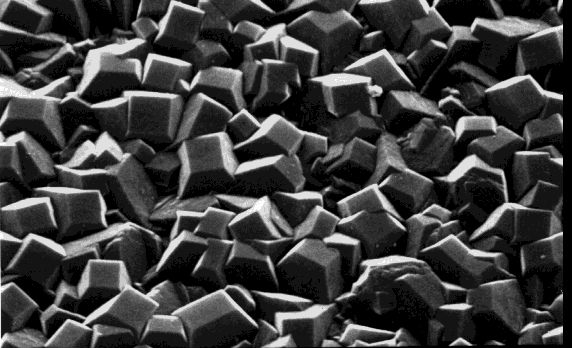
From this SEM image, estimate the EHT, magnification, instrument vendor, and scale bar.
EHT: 25 kV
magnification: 8 K X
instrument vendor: JEOL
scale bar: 5000 nm